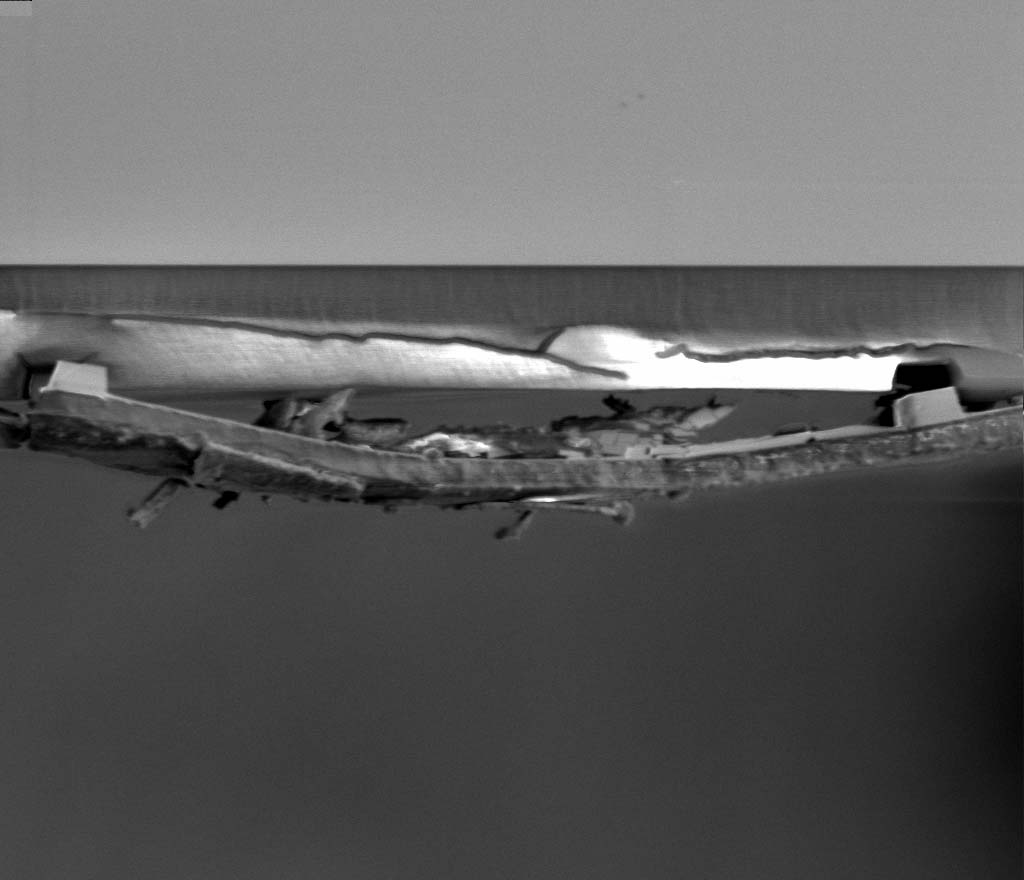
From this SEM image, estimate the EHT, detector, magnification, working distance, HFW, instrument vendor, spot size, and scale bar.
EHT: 1.5 kV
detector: ETD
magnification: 8 K X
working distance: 9.7 mm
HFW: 33.8 µm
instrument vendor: FEI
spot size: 3.6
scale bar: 5000 nm